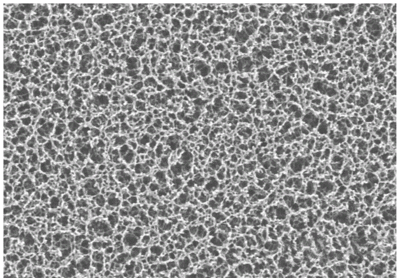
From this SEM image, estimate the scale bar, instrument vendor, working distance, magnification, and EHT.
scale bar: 20000 nm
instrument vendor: Hitachi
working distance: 15.3 mm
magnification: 2 K X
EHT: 5 kV